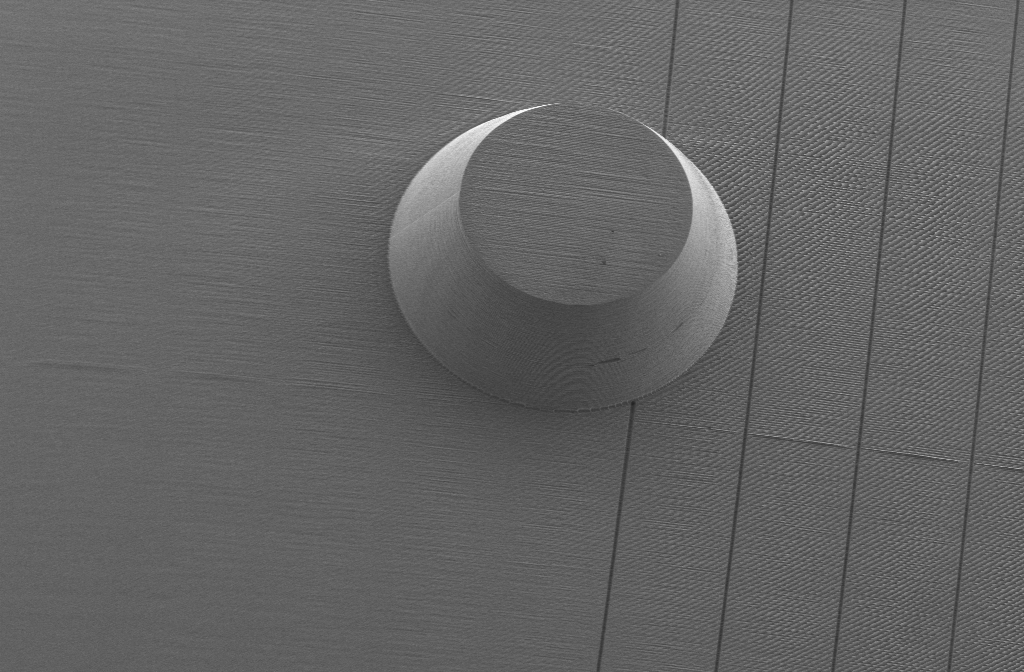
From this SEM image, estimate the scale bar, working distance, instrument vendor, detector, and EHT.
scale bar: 100000 nm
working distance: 8.6 mm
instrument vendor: Zeiss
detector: SE2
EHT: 2 kV